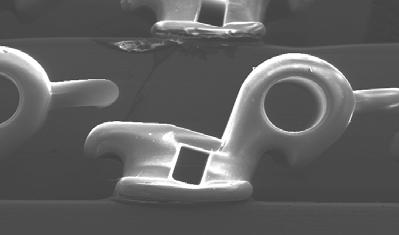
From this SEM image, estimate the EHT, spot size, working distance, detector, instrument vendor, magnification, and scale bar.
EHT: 20 kV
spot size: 7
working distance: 40 mm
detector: SE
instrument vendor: FEI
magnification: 0.035 K X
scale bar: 2000000 nm